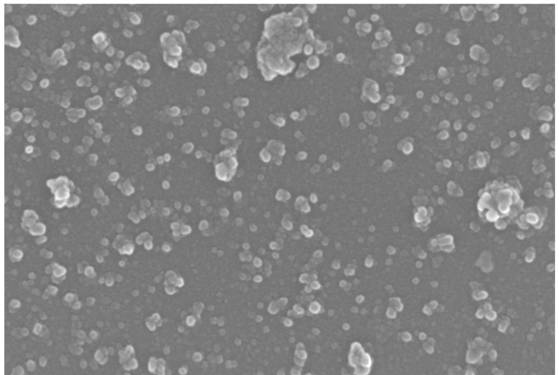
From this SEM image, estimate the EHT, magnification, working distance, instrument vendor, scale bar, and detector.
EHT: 15 kV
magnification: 45.53 K X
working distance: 6.6 mm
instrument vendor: Zeiss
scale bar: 200 nm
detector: InLens